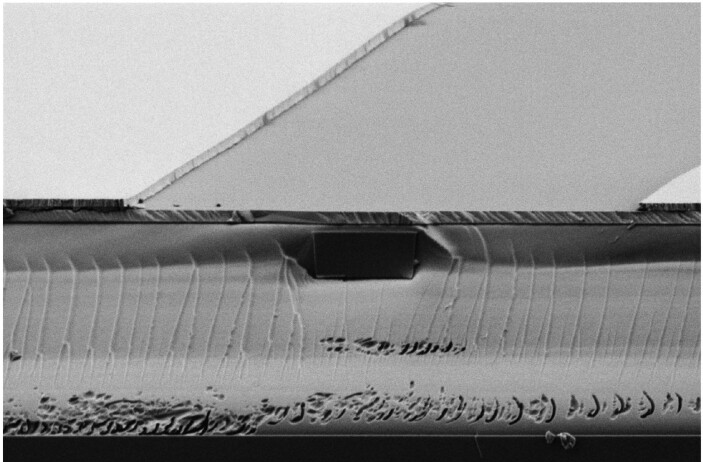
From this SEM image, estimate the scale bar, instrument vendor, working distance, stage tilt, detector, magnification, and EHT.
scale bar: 1000 nm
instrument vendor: Zeiss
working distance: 8.1 mm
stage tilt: -4°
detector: HE-SE2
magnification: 8.13 K X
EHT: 1.2 kV